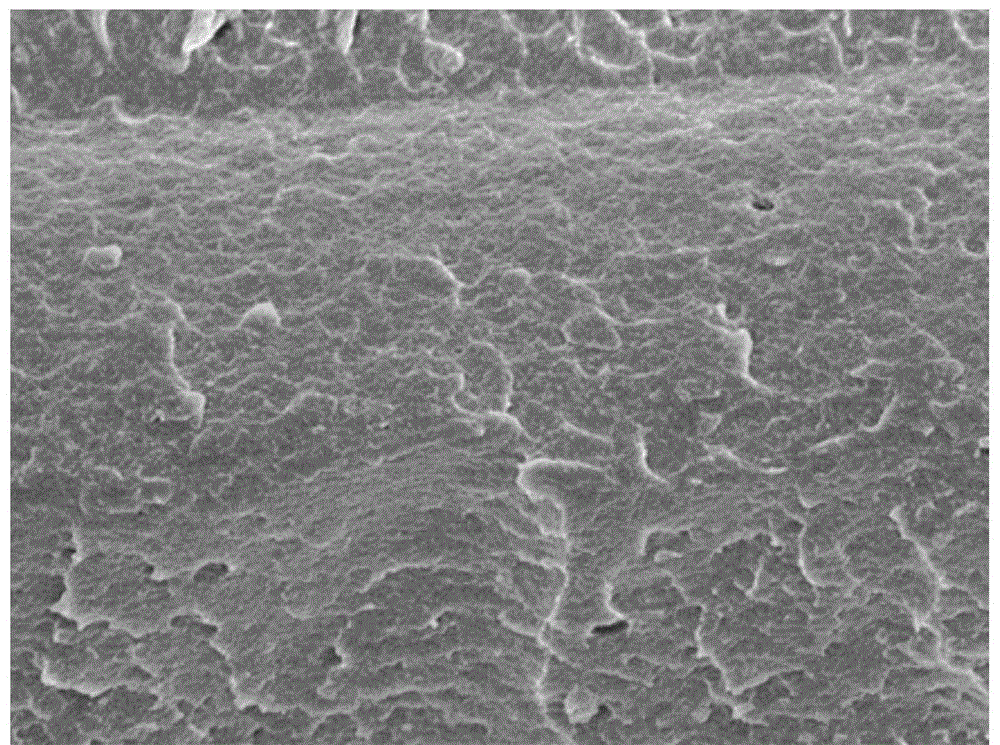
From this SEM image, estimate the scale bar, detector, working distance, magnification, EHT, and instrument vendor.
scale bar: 1000 nm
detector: SEI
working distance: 7.7 mm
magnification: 5 K X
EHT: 5 kV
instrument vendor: JEOL